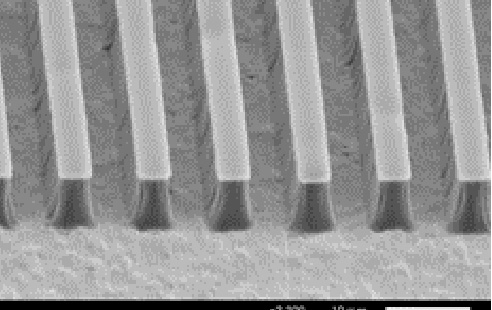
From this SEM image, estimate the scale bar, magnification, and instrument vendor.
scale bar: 10000 nm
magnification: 2.2 K X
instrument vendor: JEOL